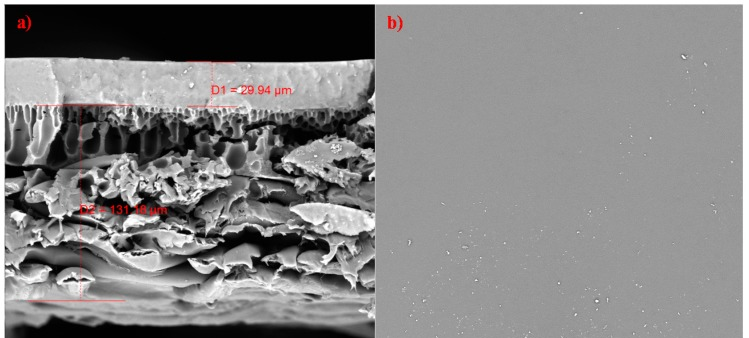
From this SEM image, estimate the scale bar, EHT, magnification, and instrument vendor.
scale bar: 200000 nm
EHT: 15 kV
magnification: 0.6 K X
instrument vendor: Tescan